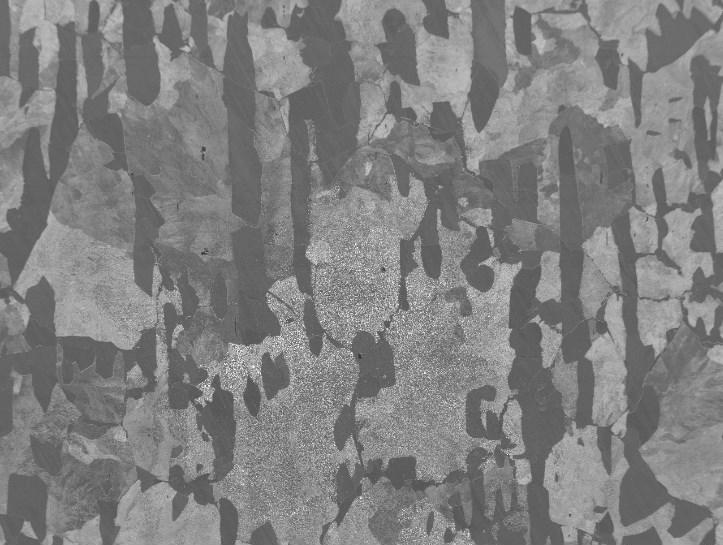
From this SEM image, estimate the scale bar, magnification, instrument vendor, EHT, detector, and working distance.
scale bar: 100000 nm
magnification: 0.14 K X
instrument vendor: JEOL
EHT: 15 kV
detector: SEI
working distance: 10 mm